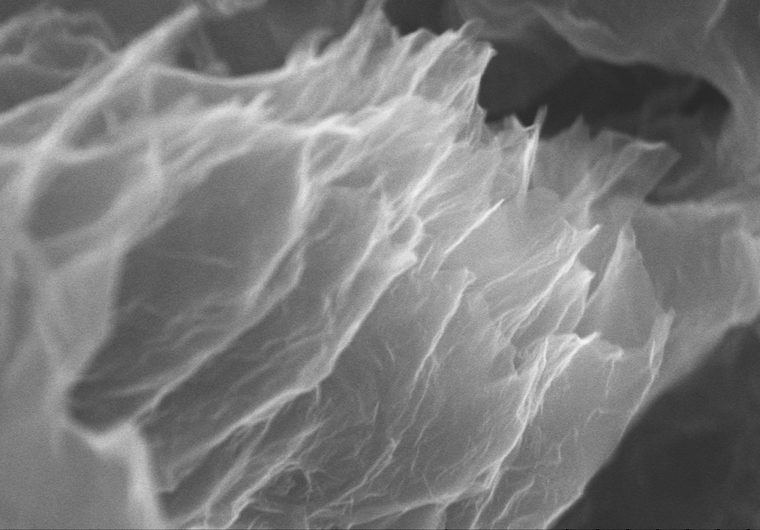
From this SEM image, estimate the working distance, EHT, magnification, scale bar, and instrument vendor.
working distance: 8.4 mm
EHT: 10 kV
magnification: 25 K X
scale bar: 2000 nm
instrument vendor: Hitachi